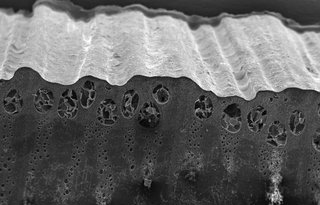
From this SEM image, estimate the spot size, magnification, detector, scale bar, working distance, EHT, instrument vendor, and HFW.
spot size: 4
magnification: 0.04 K X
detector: ETD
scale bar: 1000000 nm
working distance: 11.7 mm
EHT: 20 kV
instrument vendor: FEI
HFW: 5180 µm